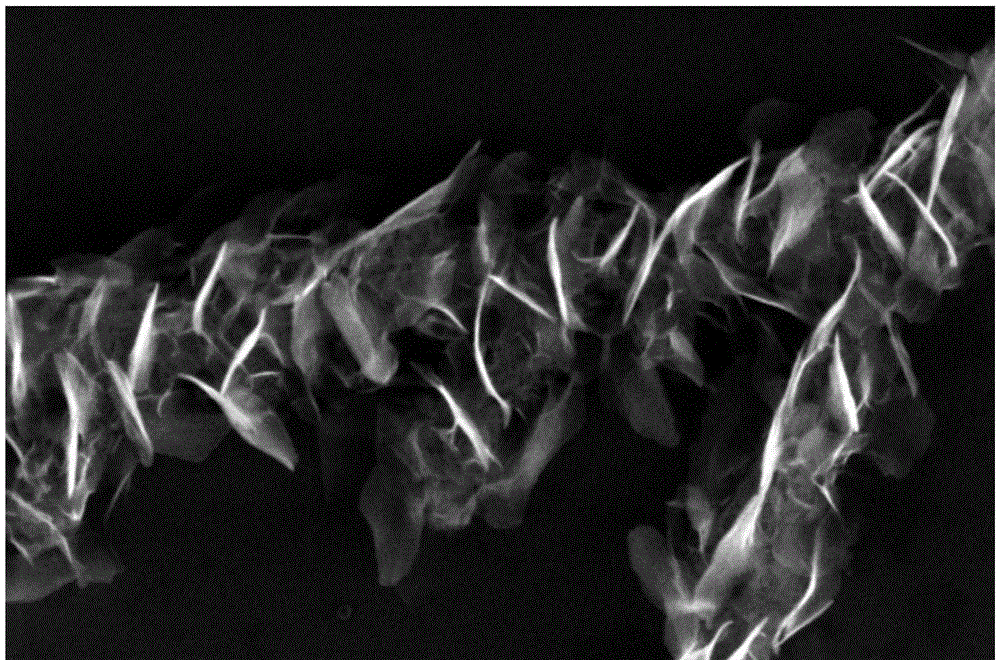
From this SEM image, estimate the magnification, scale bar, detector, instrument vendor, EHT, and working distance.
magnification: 50 K X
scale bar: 1000 nm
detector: ETD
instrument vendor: FEI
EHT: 18 kV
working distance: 4.2 mm